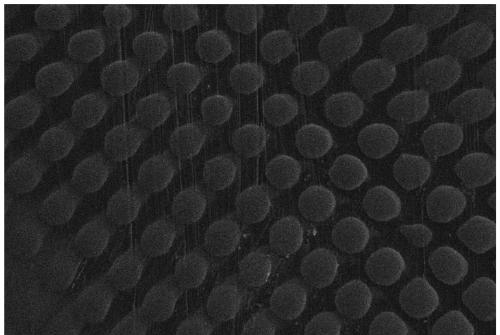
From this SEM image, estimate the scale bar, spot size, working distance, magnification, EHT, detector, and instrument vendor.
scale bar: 100000 nm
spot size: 30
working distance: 10 mm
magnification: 0.17 K X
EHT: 20 kV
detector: SEI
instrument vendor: JEOL